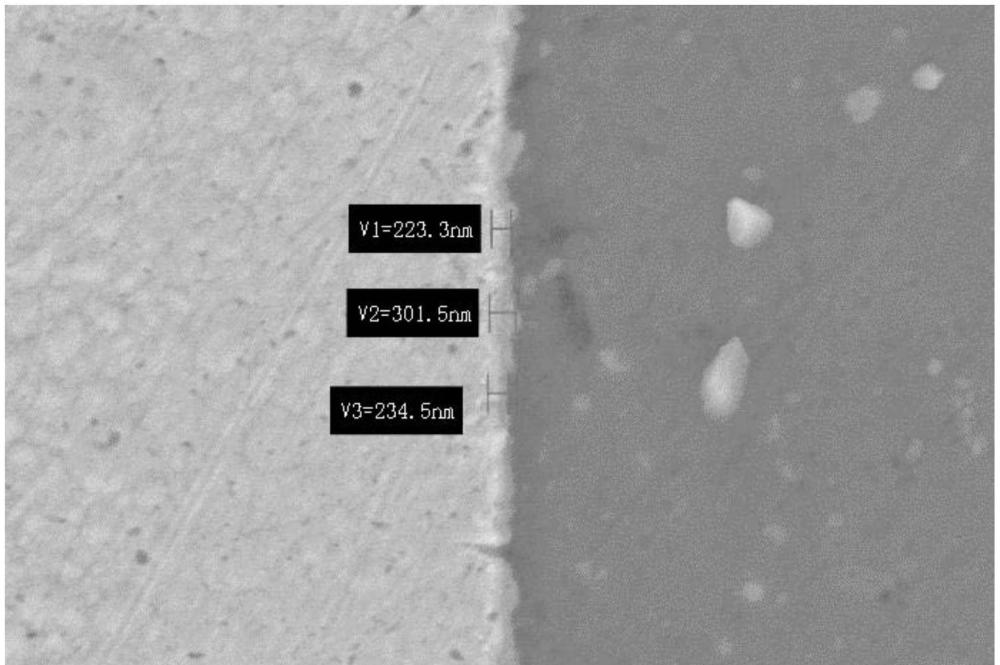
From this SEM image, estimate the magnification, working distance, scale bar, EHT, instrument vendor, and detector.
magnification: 10 K X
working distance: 7.6 mm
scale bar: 1000 nm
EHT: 10 kV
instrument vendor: Zeiss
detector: SE2